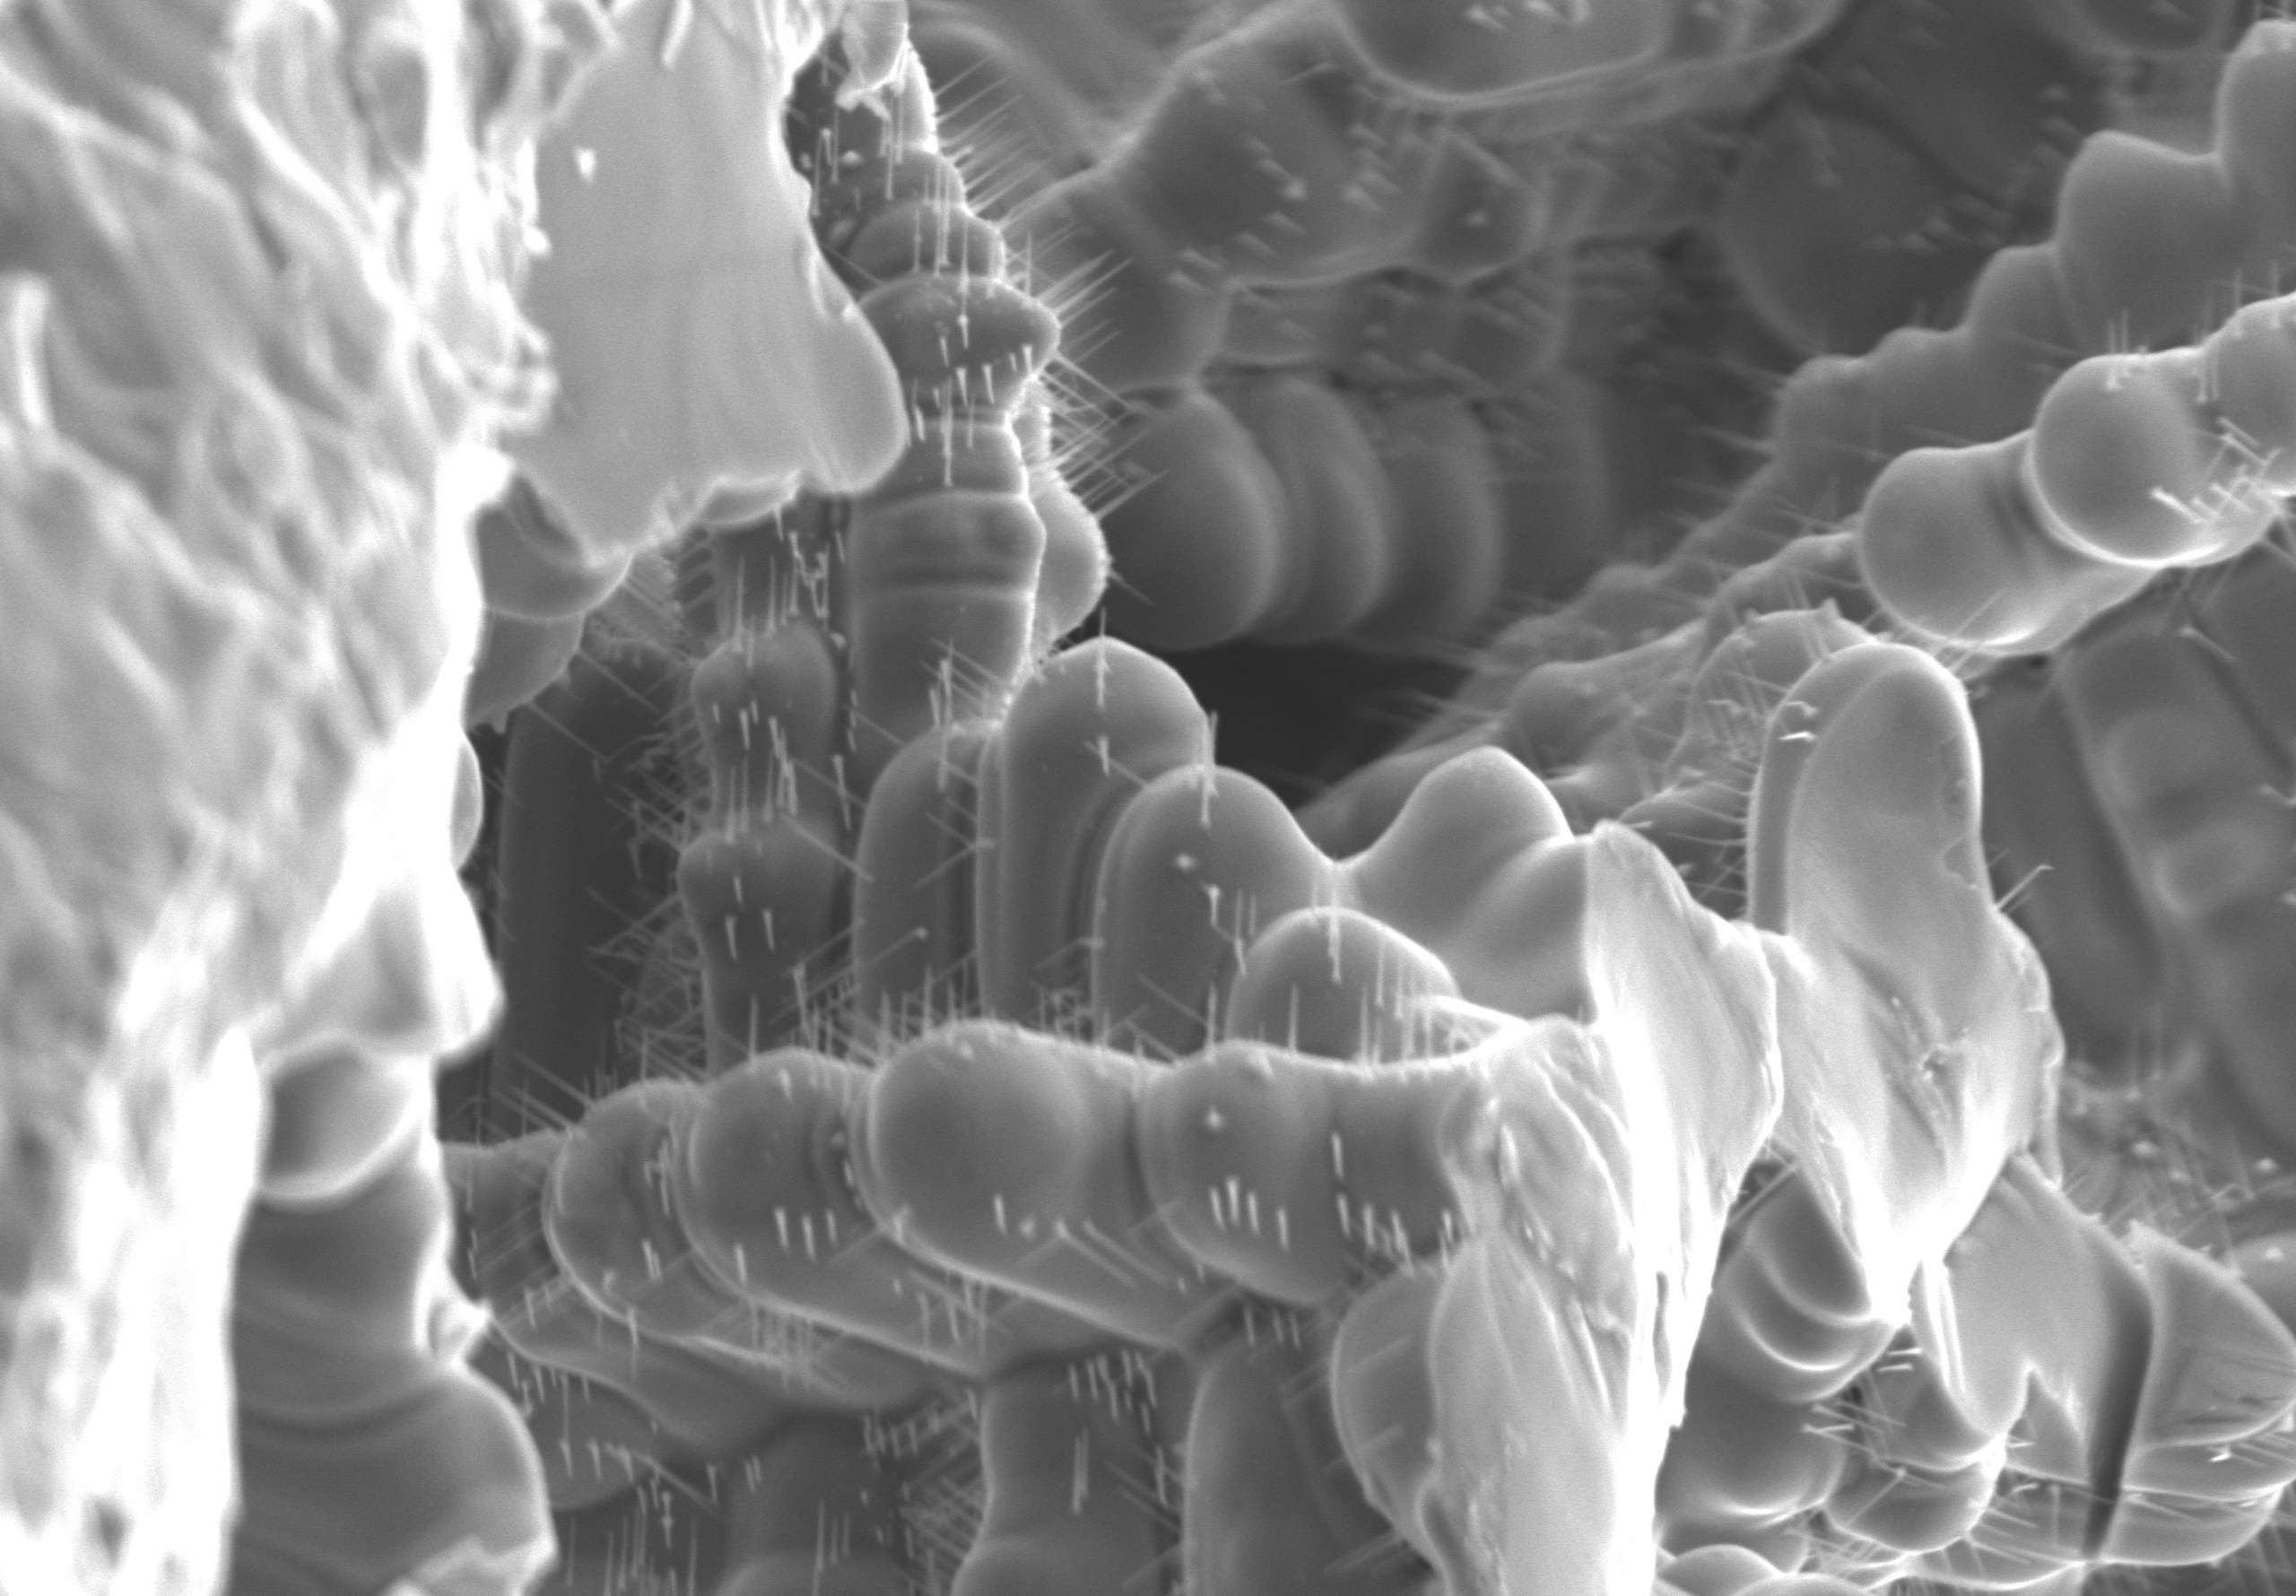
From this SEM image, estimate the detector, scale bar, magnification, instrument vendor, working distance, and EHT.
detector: SE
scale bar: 40000 nm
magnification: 1.4 K X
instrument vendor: Hitachi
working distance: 10.2 mm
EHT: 15 kV